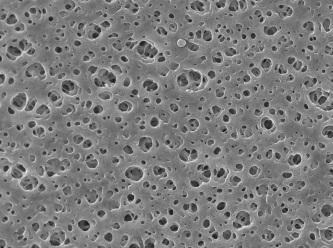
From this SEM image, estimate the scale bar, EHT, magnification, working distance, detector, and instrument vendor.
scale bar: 1000 nm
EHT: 10 kV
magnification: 10 K X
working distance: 7 mm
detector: SEI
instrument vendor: JEOL